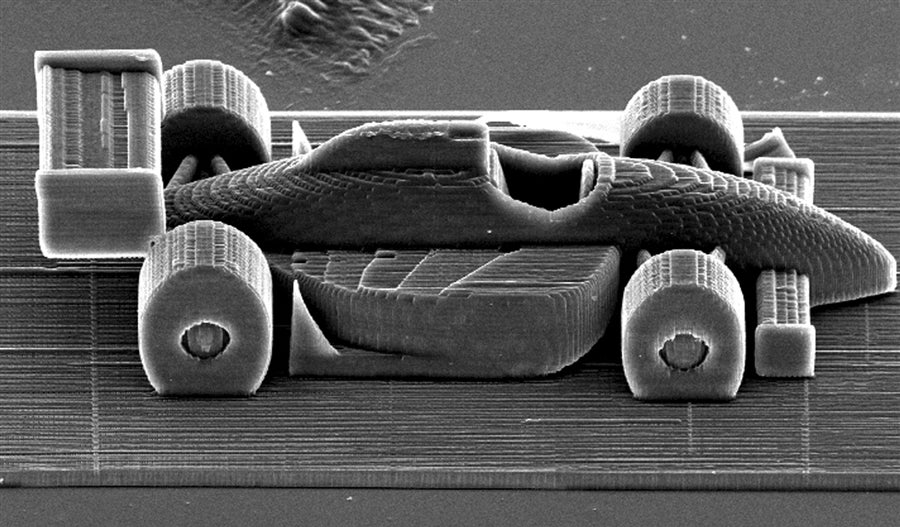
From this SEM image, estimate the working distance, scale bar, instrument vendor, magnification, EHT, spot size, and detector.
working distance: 27.2 mm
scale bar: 100000 nm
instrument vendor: FEI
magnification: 0.363 K X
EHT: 20 kV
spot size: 4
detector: SE